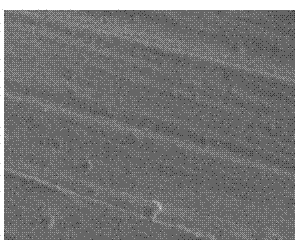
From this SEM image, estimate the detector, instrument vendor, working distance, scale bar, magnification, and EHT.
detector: SE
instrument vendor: Hitachi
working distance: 11.3 mm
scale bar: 100000 nm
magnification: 0.47 K X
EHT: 15 kV